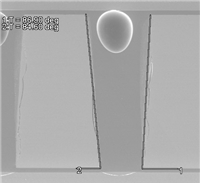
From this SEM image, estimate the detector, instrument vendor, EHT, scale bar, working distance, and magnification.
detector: SE(U)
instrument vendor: Hitachi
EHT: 10 kV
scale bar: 500000 nm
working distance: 11.2 mm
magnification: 0.1 K X